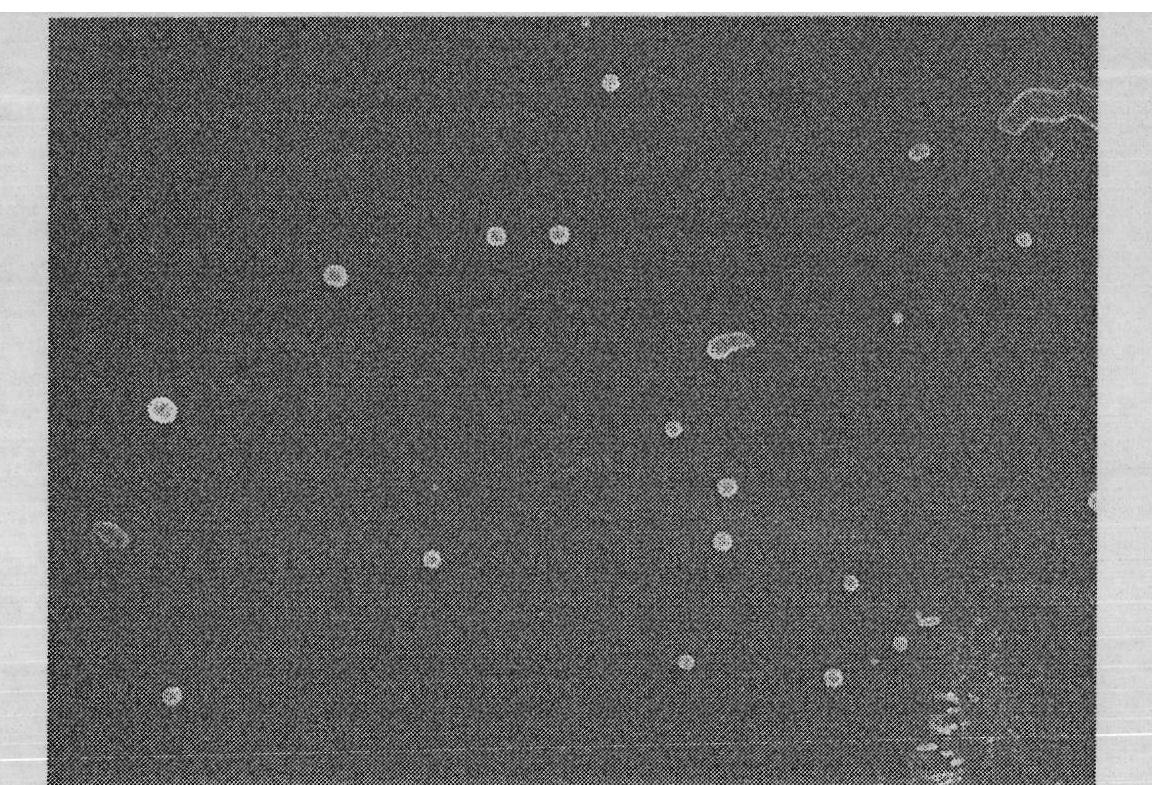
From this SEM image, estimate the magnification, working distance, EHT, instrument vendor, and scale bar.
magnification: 10 K X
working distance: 8.4 mm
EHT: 8 kV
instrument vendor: JEOL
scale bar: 1000 nm